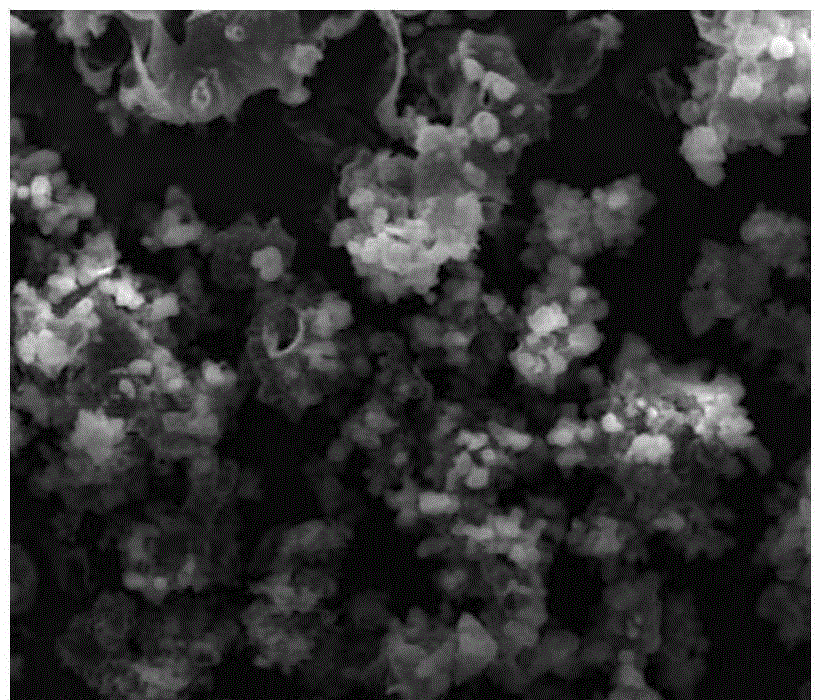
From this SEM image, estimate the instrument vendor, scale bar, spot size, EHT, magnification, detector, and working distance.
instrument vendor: FEI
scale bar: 100000 nm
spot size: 3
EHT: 30 kV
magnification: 1 K X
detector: ETD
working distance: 21.3 mm